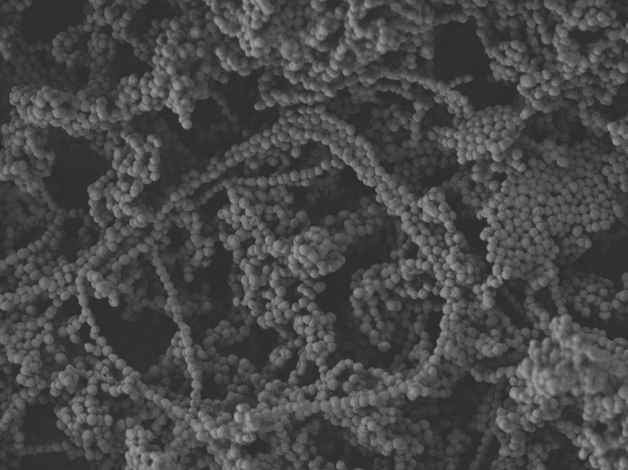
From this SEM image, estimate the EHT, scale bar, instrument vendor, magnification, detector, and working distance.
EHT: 5 kV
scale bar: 10000 nm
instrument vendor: JEOL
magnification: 2 K X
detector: LEI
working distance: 8 mm